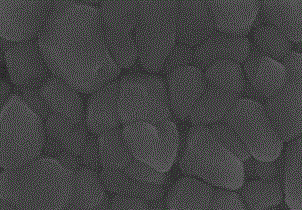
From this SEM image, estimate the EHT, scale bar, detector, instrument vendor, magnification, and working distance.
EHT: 7 kV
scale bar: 500 nm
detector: SE(U)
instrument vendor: Hitachi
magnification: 100 K X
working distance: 3.9 mm